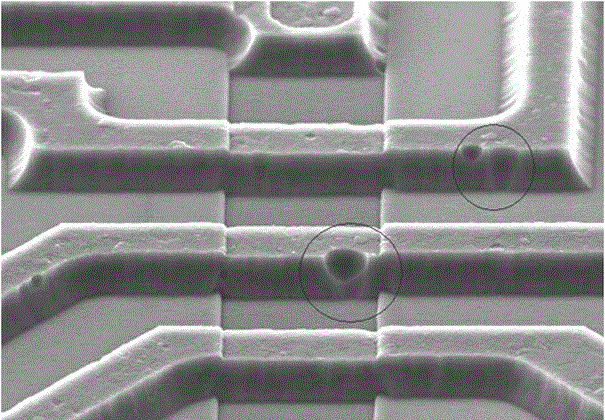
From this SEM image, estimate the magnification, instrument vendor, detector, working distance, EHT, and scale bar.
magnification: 2 K X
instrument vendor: Hitachi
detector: SE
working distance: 14.7 mm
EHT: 15 kV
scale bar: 20000 nm